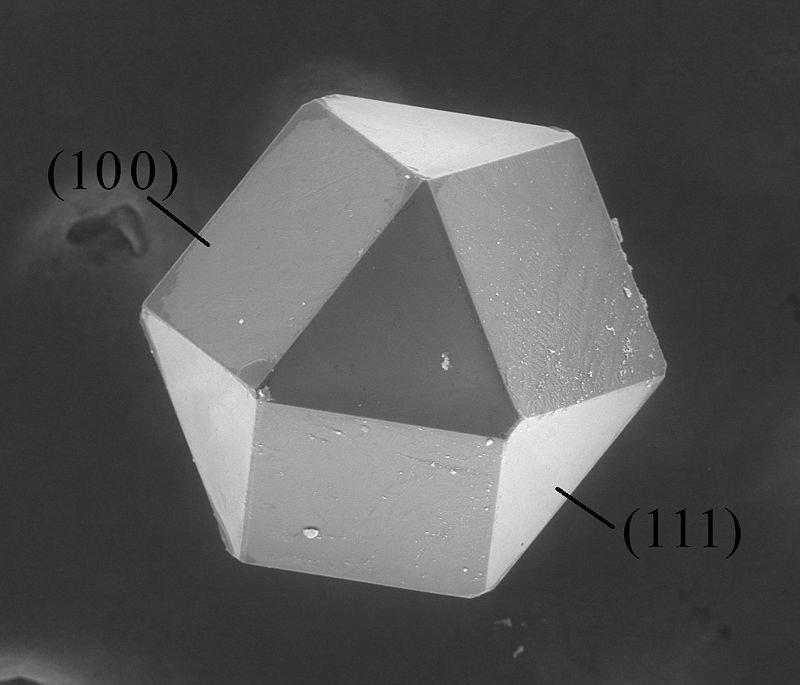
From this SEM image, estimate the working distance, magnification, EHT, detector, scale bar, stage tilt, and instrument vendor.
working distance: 13.1 mm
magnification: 0.4 K X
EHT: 20 kV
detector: LFD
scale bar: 200000 nm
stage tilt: -0°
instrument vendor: FEI